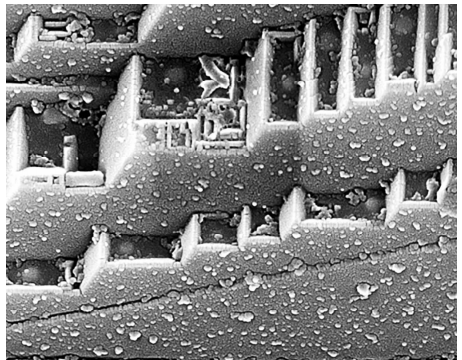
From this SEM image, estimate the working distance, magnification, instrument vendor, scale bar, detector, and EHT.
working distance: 5.4 mm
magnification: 100 K X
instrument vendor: FEI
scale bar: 500 nm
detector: TLD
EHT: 18 kV